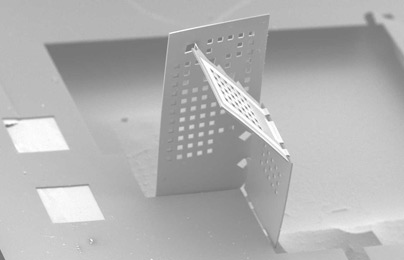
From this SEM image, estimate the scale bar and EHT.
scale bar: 500000 nm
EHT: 10 kV